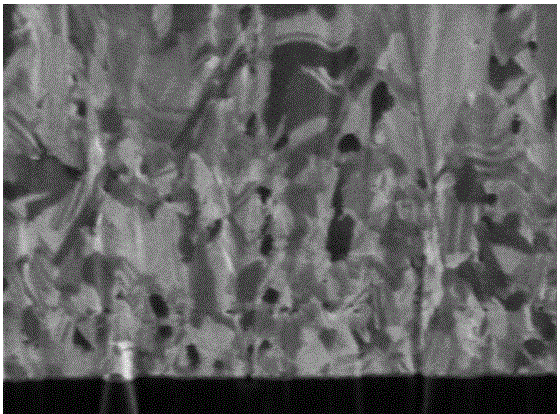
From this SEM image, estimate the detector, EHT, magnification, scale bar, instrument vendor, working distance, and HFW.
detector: SED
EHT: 30 kV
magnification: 80 K X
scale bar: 500 nm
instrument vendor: FEI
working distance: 18 mm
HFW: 3.8 µm